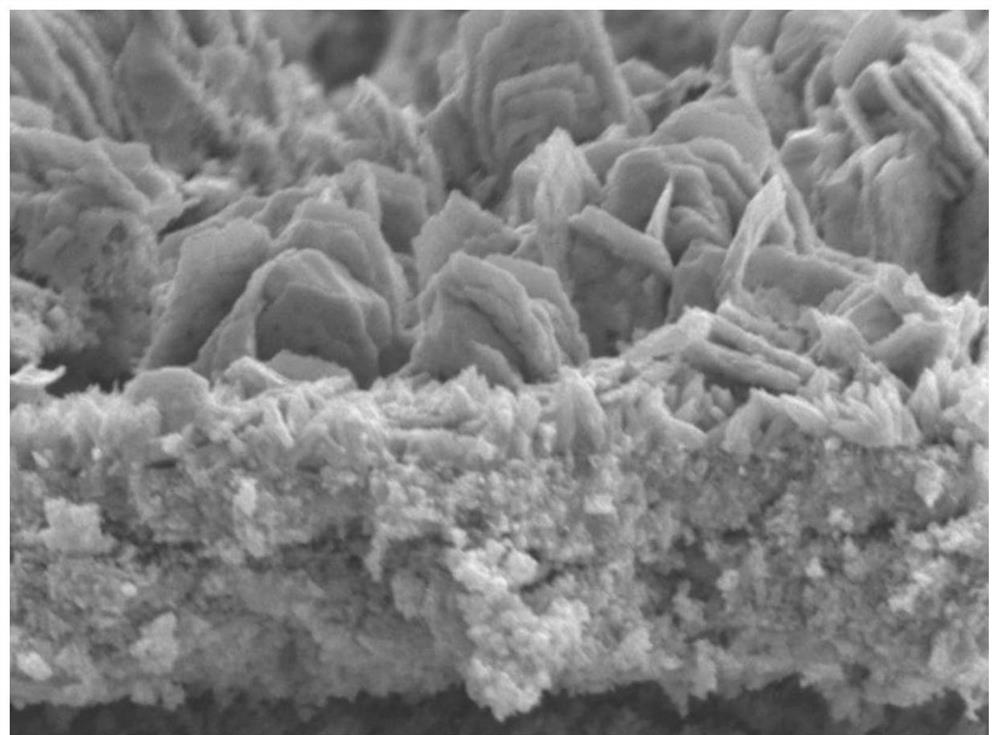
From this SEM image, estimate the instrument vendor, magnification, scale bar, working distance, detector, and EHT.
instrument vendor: JEOL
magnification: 25 K X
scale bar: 1000 nm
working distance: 10.1 mm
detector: LED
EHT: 5 kV